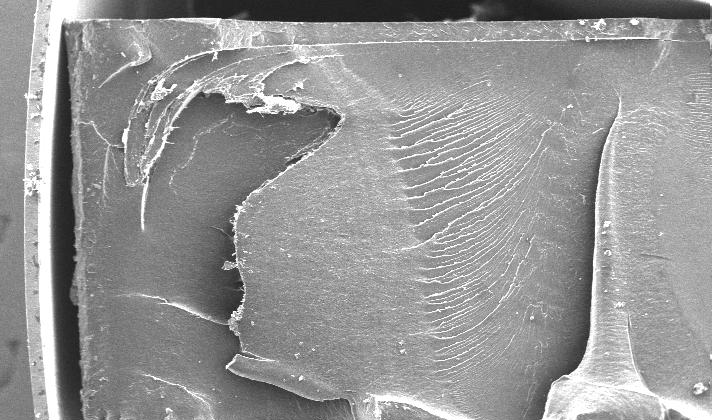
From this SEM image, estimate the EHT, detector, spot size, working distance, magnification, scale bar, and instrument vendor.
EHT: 20 kV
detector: SE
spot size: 5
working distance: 9.4 mm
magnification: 0.052 K X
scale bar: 1000000 nm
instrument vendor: FEI